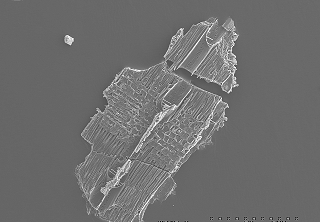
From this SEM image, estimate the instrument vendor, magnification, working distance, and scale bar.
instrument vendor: JEOL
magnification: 0.07 K X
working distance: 15 mm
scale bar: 500000 nm